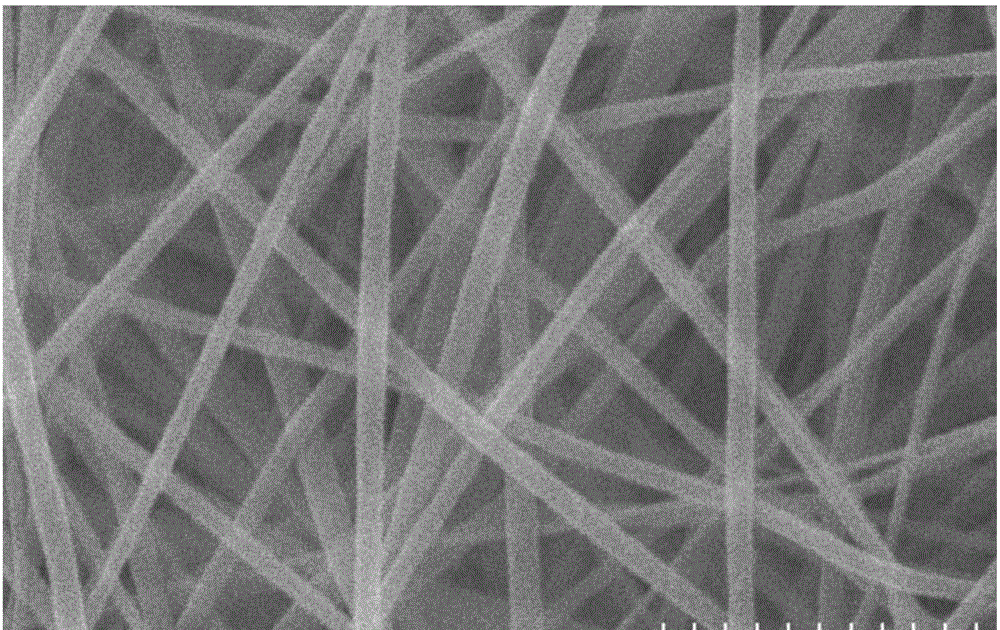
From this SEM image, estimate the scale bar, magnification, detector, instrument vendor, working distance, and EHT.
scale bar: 2000 nm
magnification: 20 K X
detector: SE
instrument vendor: Hitachi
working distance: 11.5 mm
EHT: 20 kV